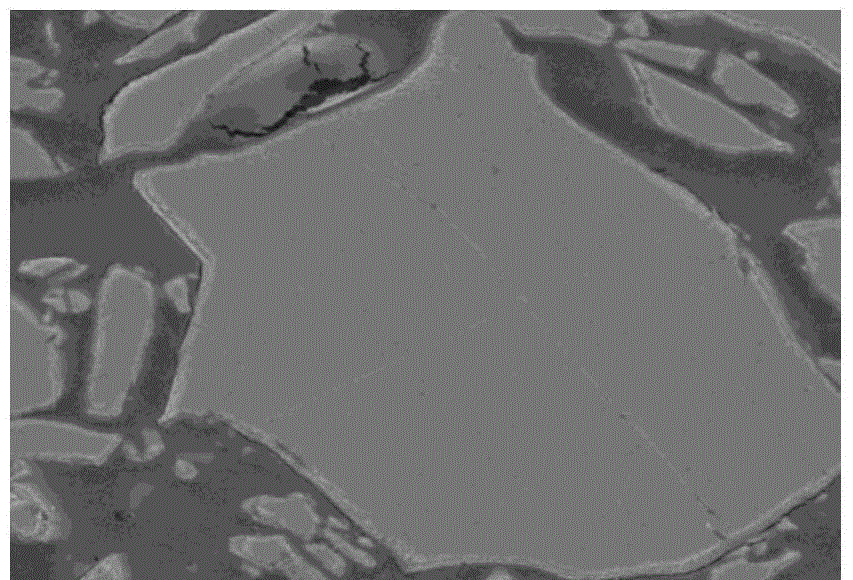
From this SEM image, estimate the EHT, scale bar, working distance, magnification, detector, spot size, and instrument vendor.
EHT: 10 kV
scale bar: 5000 nm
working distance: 11.4 mm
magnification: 10 K X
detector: ETD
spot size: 3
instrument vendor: FEI